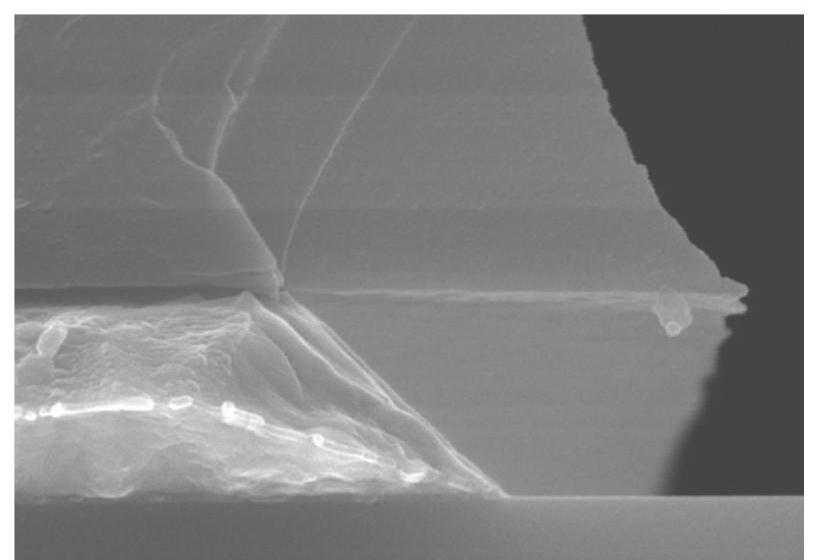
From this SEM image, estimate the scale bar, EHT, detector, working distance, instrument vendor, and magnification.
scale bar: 1000 nm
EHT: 15 kV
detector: SE(UL)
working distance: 11.9 mm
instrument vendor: Hitachi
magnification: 50 K X